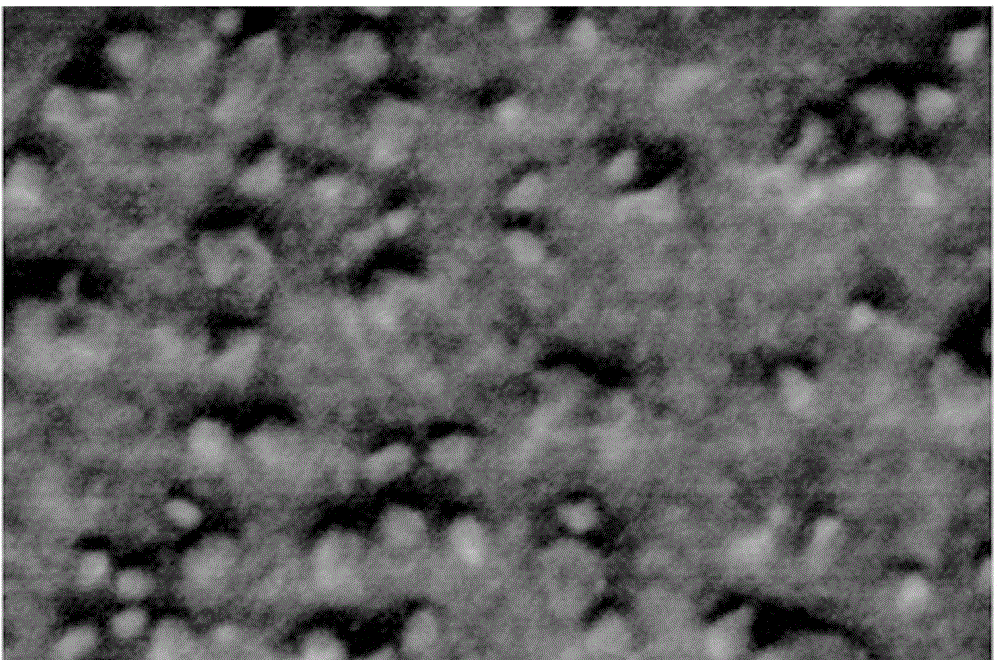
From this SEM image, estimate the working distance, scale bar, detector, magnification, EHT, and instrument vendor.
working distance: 1.9 mm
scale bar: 500 nm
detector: SE(U, LA0)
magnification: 100 K X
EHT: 1 kV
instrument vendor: Hitachi